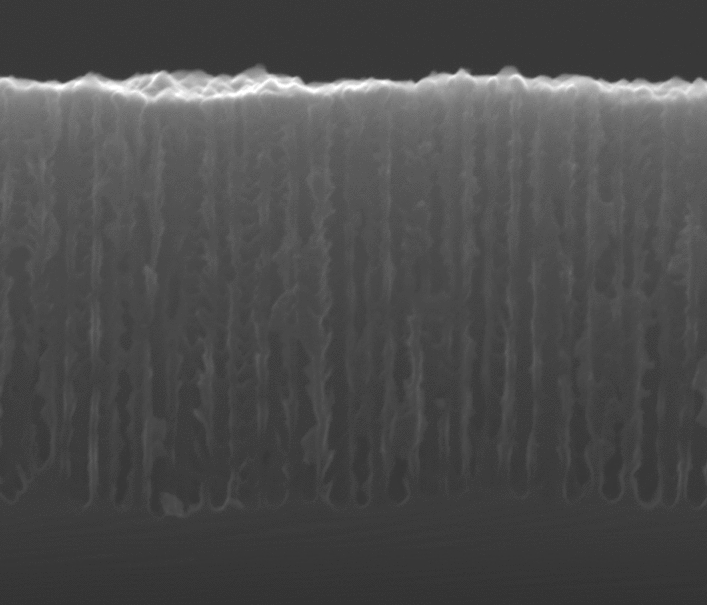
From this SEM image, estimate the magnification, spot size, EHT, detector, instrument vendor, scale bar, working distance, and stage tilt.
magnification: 100 K X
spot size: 3.5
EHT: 20 kV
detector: ETD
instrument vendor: FEI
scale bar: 500 nm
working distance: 9.5 mm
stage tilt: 0°